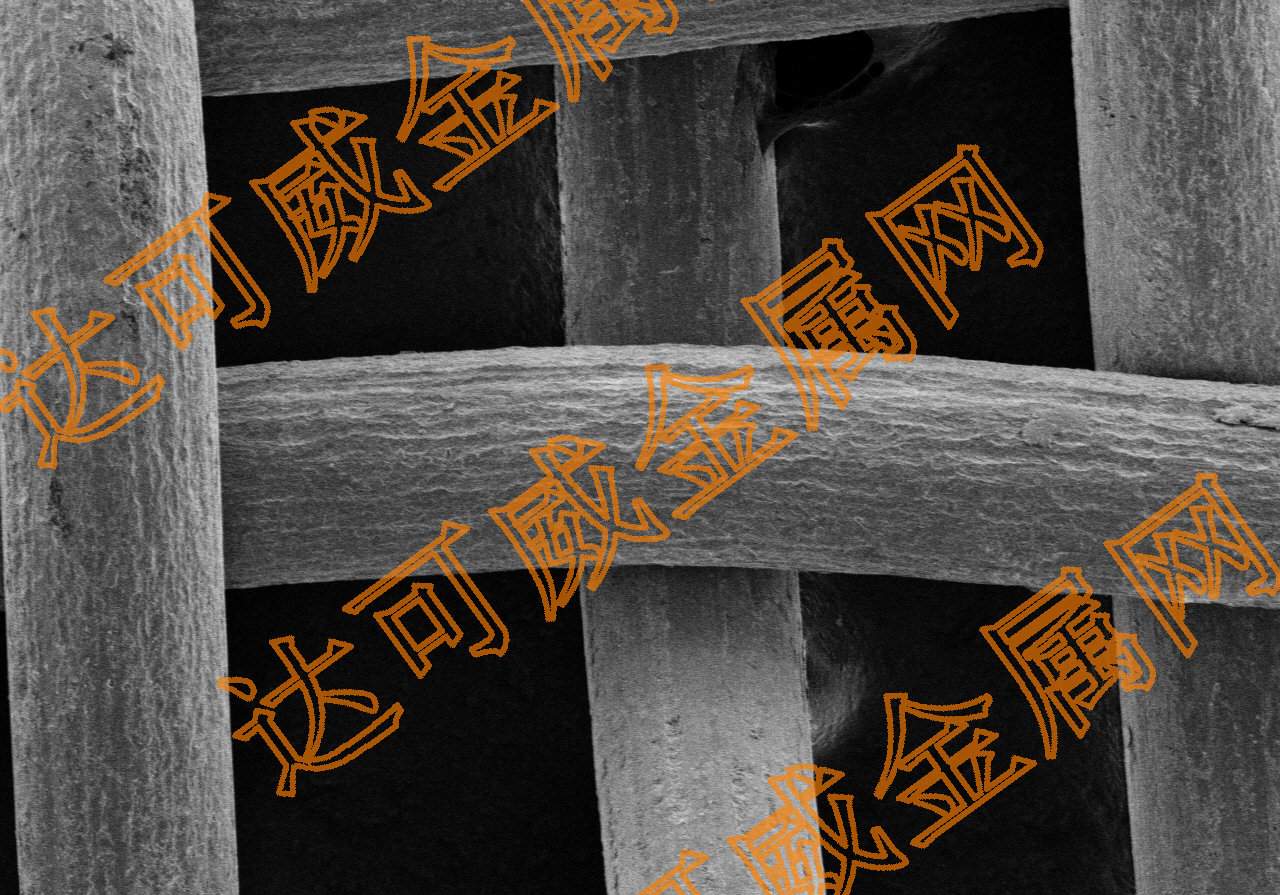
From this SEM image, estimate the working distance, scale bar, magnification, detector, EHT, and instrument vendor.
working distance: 7.3 mm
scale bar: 200000 nm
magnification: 0.22 K X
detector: SE(M)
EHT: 5 kV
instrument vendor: Hitachi